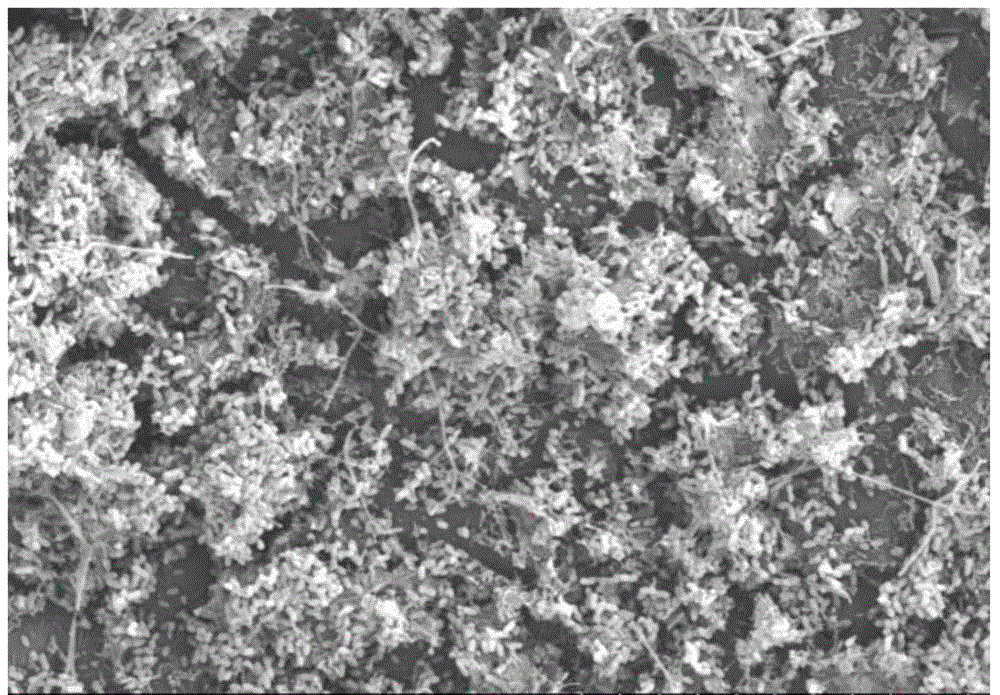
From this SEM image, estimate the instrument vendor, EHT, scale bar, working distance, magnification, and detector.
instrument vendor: Hitachi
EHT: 15 kV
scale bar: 50000 nm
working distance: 13 mm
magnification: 1 K X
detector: SE(M)